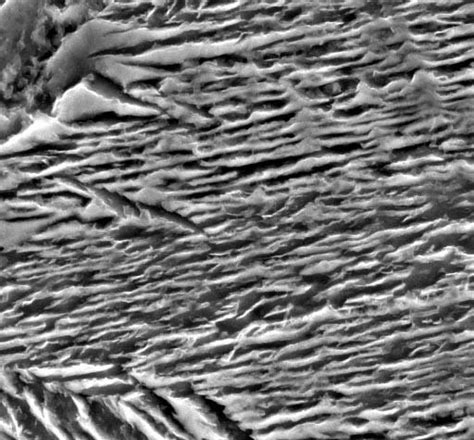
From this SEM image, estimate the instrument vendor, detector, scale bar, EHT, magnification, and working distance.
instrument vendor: JEOL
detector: SEI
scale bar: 1000 nm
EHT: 10 kV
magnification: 20 K X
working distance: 5.7 mm